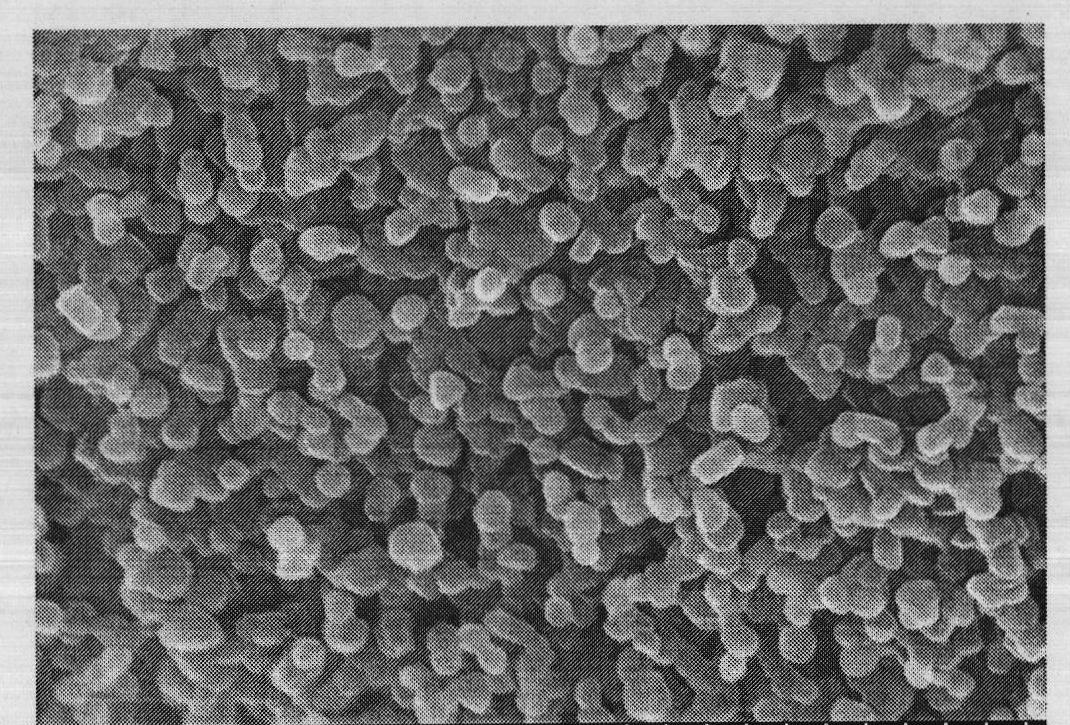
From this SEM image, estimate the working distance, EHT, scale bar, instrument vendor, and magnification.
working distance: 4.2 mm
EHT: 15 kV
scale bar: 1000 nm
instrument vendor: Hitachi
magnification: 50 K X